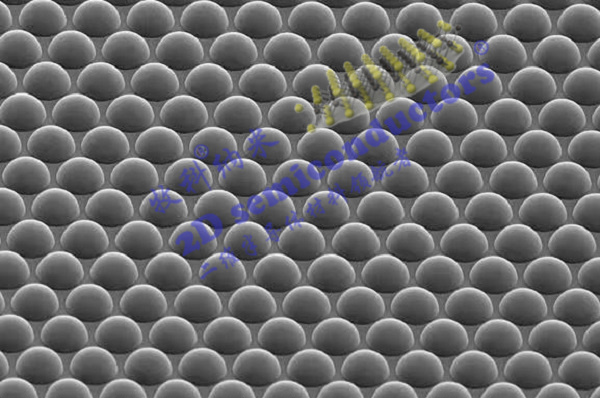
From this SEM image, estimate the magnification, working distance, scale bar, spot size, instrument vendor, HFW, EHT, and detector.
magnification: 0.5 K X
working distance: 11.8 mm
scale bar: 100000 nm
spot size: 3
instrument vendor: FEI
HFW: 254 µm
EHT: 10 kV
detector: ETD